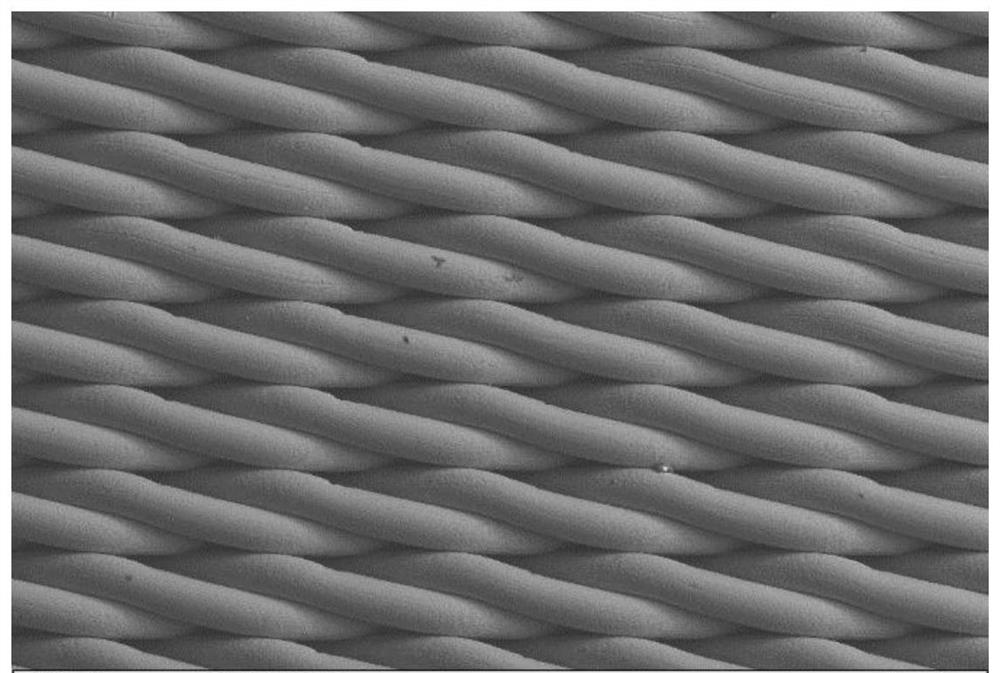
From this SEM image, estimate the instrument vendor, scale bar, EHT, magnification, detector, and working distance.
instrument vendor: Zeiss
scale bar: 100000 nm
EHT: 15 kV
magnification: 0.1 K X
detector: SE2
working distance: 10.3 mm